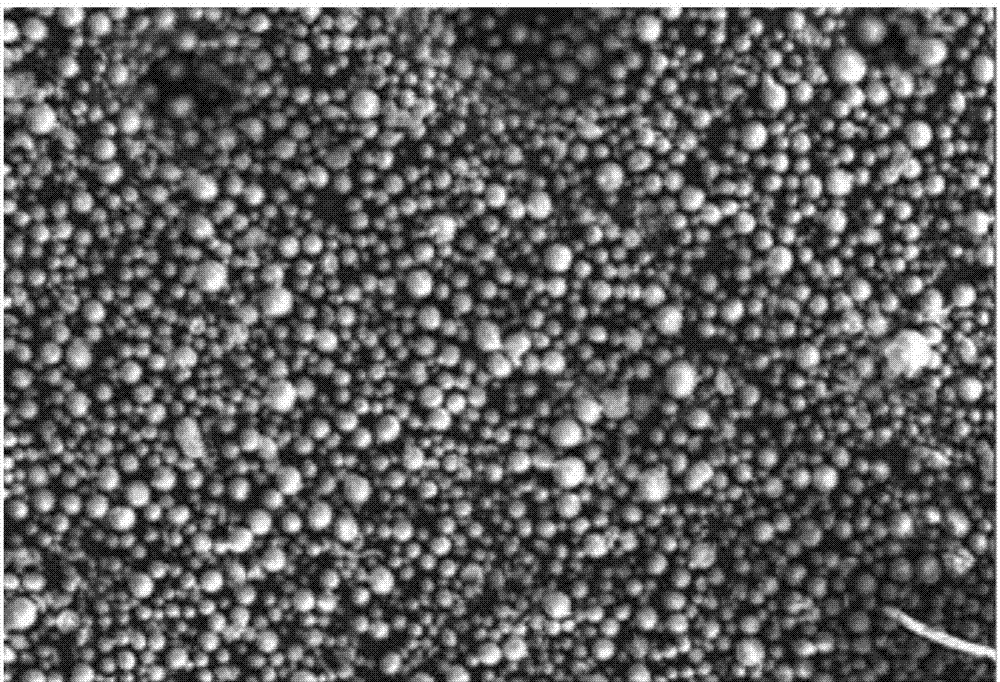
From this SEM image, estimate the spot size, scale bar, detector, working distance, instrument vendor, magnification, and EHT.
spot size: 5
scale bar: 200000 nm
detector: SE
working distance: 11.5 mm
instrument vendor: FEI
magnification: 0.098 K X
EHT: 10 kV